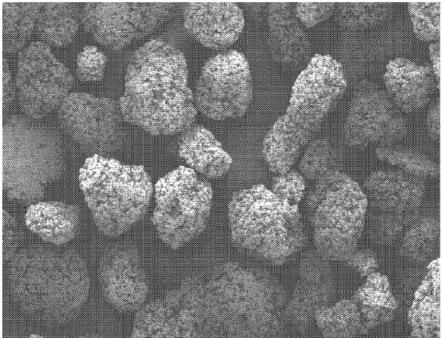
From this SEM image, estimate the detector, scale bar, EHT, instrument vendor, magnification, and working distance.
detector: SE(M)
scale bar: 500000 nm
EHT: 5 kV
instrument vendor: Hitachi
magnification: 0.1 K X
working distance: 12.8 mm